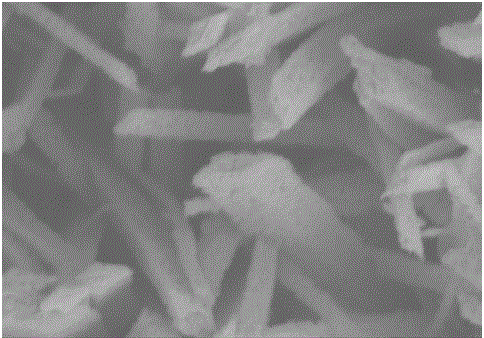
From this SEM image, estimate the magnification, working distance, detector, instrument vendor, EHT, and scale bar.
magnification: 50 K X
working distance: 9.3 mm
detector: SE(M)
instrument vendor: Hitachi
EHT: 5 kV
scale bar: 1000 nm